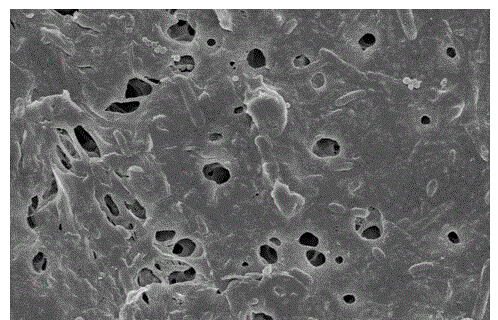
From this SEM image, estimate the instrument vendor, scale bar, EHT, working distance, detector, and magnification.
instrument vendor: Hitachi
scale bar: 10000 nm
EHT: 5 kV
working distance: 6.3 mm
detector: SE(M)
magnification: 5 K X